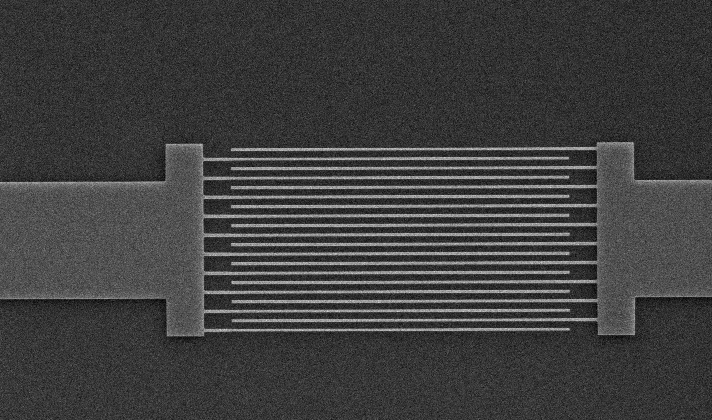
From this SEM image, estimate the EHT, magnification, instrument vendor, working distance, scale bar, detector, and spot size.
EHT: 15 kV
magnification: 2.301 K X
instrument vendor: FEI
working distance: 5.1 mm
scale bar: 10000 nm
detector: SE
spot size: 3